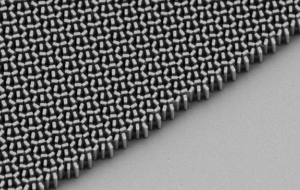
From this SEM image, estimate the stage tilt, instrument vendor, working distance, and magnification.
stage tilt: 34.2°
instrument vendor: Zeiss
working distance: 6.1 mm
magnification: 22.81 K X